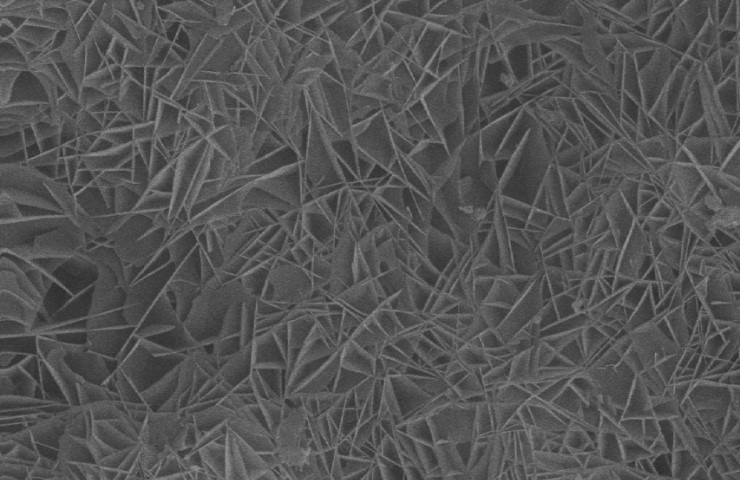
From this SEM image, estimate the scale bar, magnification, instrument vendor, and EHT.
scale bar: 2000 nm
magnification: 5 K X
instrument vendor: JEOL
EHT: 20 kV